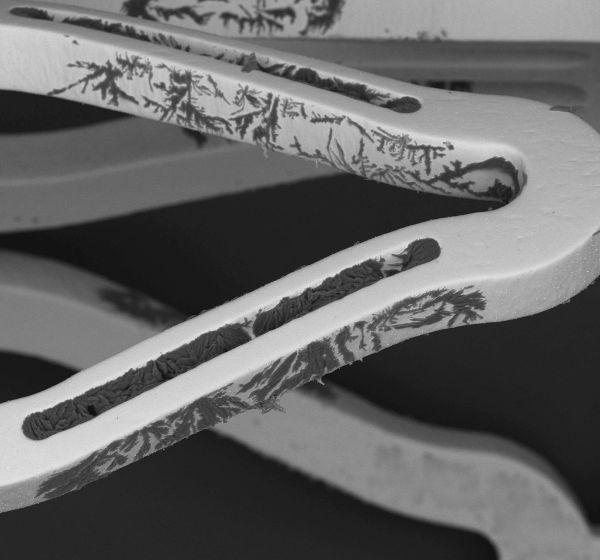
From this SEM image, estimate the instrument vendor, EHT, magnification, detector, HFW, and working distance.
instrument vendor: other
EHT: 15 kV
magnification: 0.125 K X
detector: BSD Full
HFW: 1000 µm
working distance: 10.557 mm